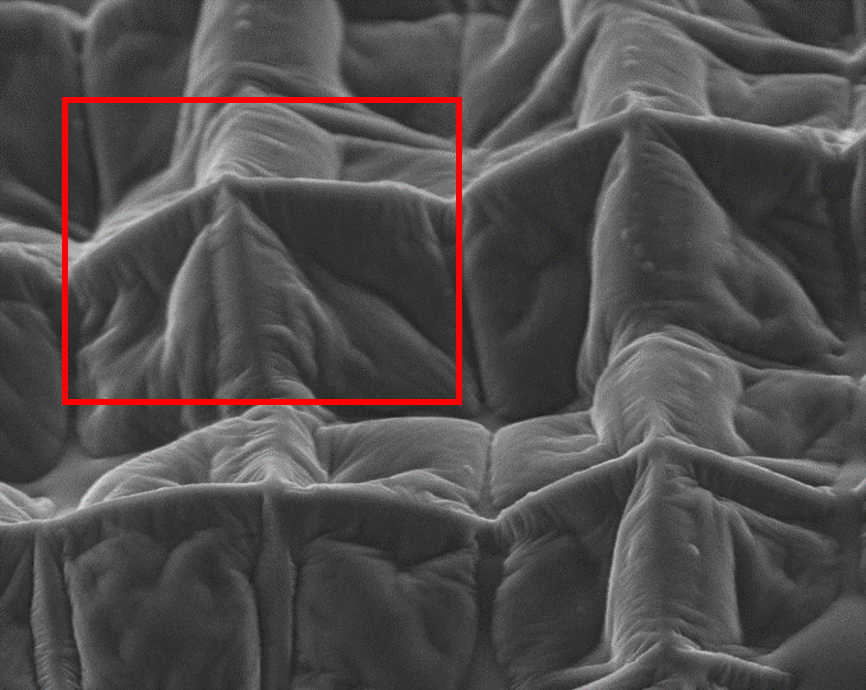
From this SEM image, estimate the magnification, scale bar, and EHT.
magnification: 1.5 K X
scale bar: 20000 nm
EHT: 10 kV